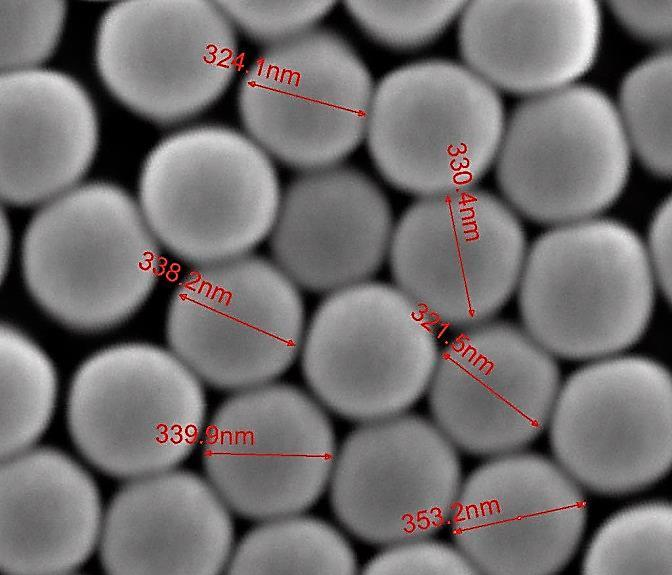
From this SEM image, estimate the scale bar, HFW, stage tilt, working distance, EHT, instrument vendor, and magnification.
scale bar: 500 nm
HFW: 1.8 µm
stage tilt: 0°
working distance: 10 mm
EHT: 25 kV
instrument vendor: FEI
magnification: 150 K X